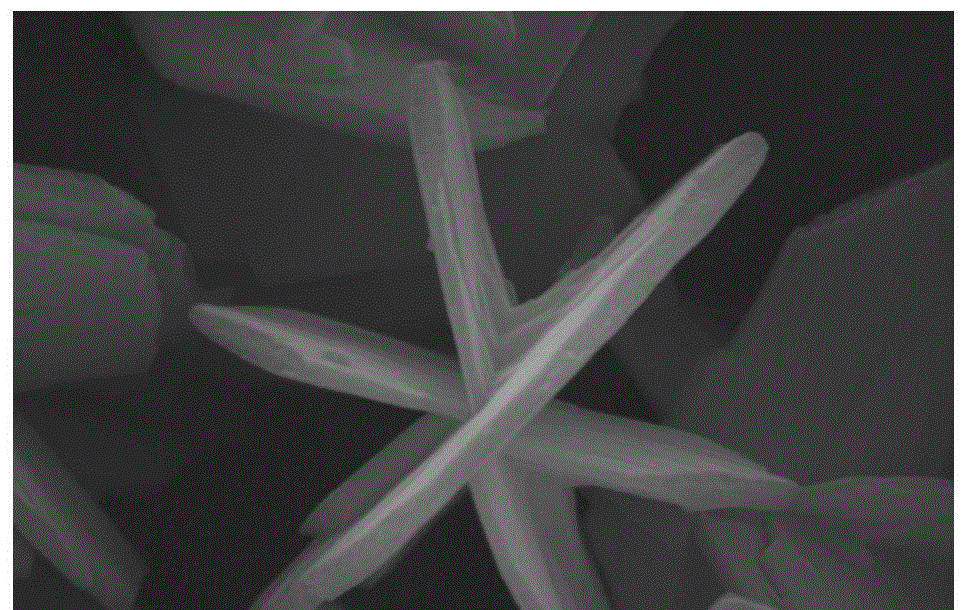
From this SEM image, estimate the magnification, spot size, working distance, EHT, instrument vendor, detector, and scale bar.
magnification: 31.621 K X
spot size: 3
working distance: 6 mm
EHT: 20 kV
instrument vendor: FEI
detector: SE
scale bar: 1000 nm